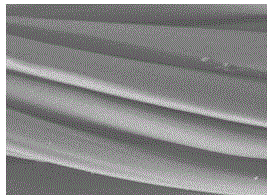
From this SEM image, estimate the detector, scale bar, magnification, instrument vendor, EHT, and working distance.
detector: LEI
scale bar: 10000 nm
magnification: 2 K X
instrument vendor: JEOL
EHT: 10 kV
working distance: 8.2 mm